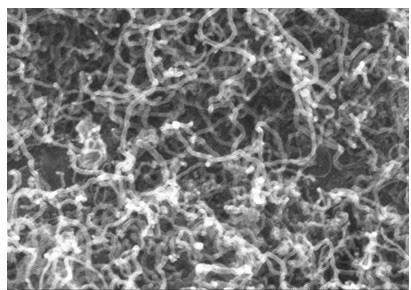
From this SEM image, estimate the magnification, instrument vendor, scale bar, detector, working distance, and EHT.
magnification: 100 K X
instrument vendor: Hitachi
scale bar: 500 nm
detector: SE(M)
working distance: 7.4 mm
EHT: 10 kV